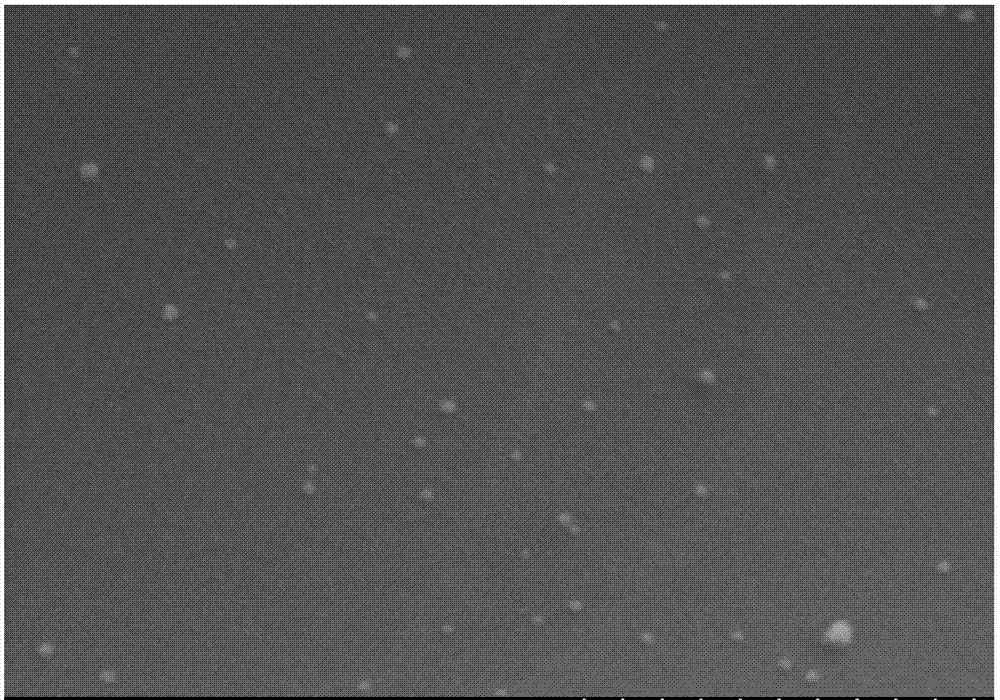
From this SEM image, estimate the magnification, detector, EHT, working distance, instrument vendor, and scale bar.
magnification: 50 K X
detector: SE(M)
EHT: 5 kV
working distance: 9.5 mm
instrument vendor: Hitachi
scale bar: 1000 nm